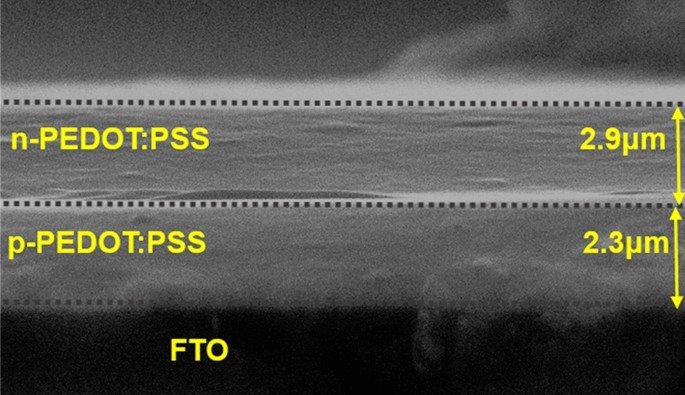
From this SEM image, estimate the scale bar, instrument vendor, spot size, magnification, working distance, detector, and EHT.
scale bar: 5000 nm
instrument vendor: JEOL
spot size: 30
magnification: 5 K X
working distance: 12 mm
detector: SEI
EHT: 20 kV